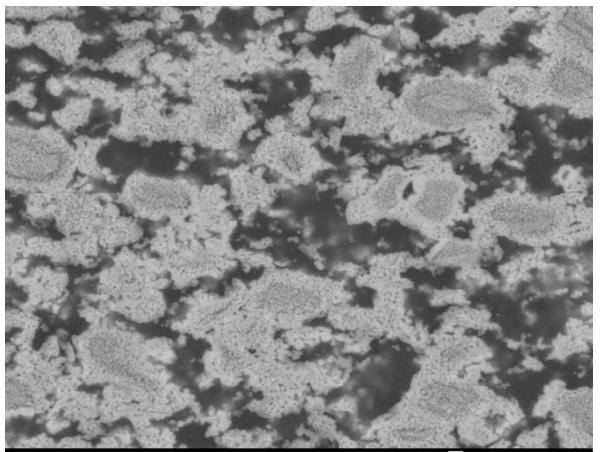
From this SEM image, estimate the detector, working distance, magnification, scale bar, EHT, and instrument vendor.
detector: COMPO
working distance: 10 mm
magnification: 3 K X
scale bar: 1000 nm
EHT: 20 kV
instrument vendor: JEOL